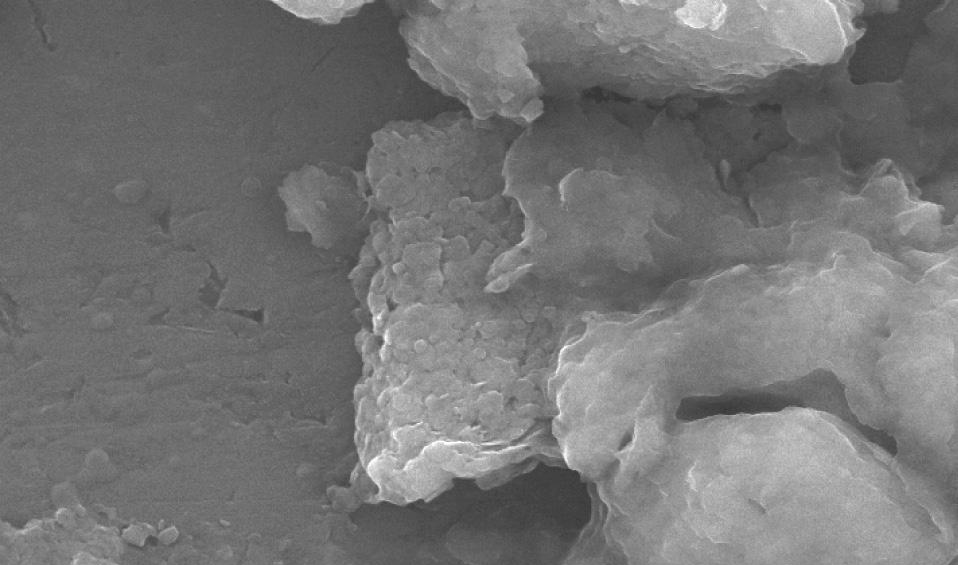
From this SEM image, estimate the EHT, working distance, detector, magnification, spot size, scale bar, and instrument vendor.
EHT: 20 kV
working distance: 11.4 mm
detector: SE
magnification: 10 K X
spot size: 3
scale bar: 2000 nm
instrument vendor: FEI